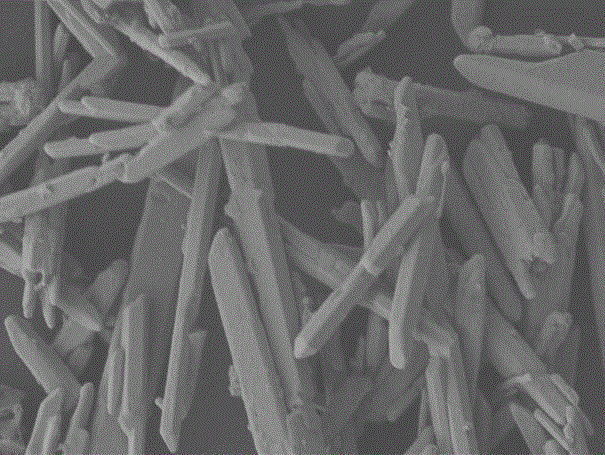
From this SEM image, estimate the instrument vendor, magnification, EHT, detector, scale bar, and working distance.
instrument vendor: JEOL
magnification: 4 K X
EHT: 5 kV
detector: LEI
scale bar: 1000 nm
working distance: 8.2 mm